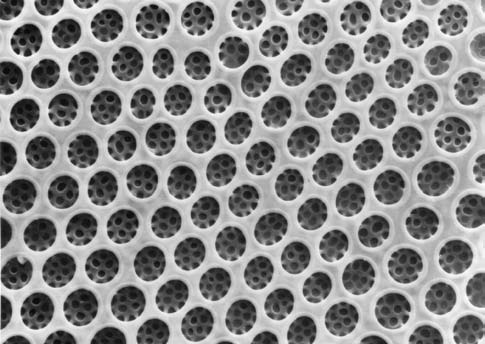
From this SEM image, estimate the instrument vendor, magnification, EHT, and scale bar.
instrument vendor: Hitachi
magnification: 5 K X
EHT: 10 kV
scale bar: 6000 nm